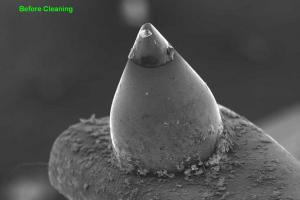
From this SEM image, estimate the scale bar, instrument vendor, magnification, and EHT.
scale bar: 100000 nm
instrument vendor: JEOL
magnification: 0.12 K X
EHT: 15 kV